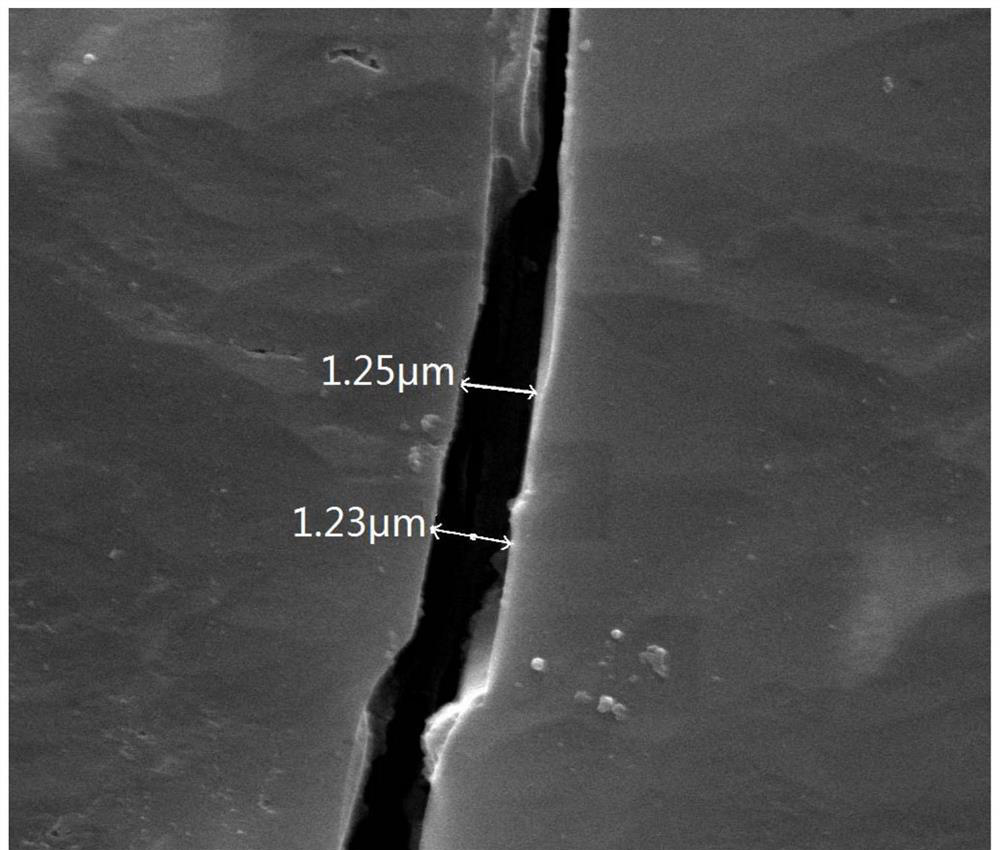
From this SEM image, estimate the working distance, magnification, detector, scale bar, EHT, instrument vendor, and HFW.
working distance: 11.8 mm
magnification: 20 K X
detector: ETD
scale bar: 5000 nm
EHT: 30 kV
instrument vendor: FEI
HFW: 14.9 µm